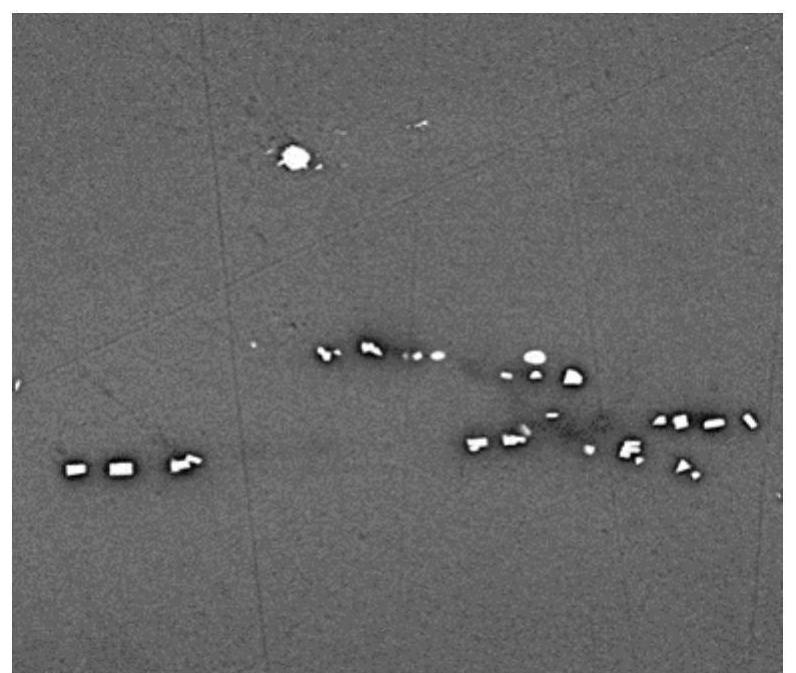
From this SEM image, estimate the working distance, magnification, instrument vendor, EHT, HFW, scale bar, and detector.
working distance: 12.9 mm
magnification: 2 K X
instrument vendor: FEI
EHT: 10 kV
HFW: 74.6 µm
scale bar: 20000 nm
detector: BSED